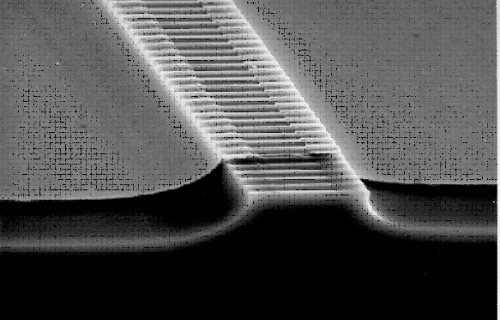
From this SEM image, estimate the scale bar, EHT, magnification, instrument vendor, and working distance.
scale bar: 10000 nm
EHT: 35 kV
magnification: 1.8 K X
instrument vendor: JEOL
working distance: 24 mm